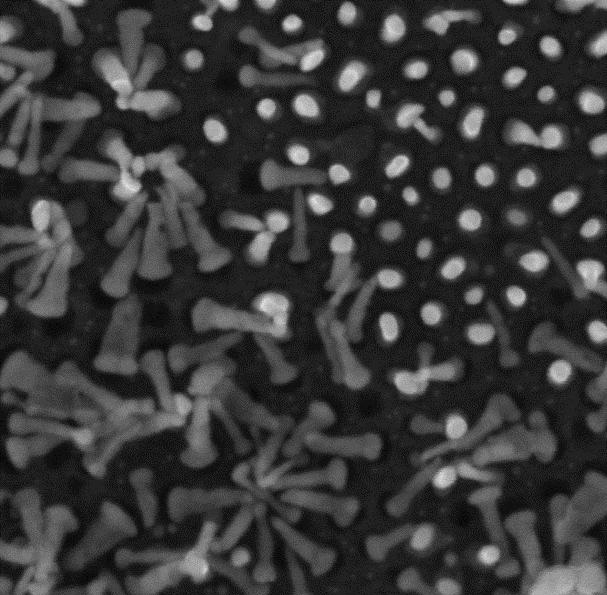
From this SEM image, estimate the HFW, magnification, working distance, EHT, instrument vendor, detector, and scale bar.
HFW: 1.156 µm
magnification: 250 K X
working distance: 3.017 mm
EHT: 15 kV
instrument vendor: Tescan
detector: InBeam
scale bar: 200 nm